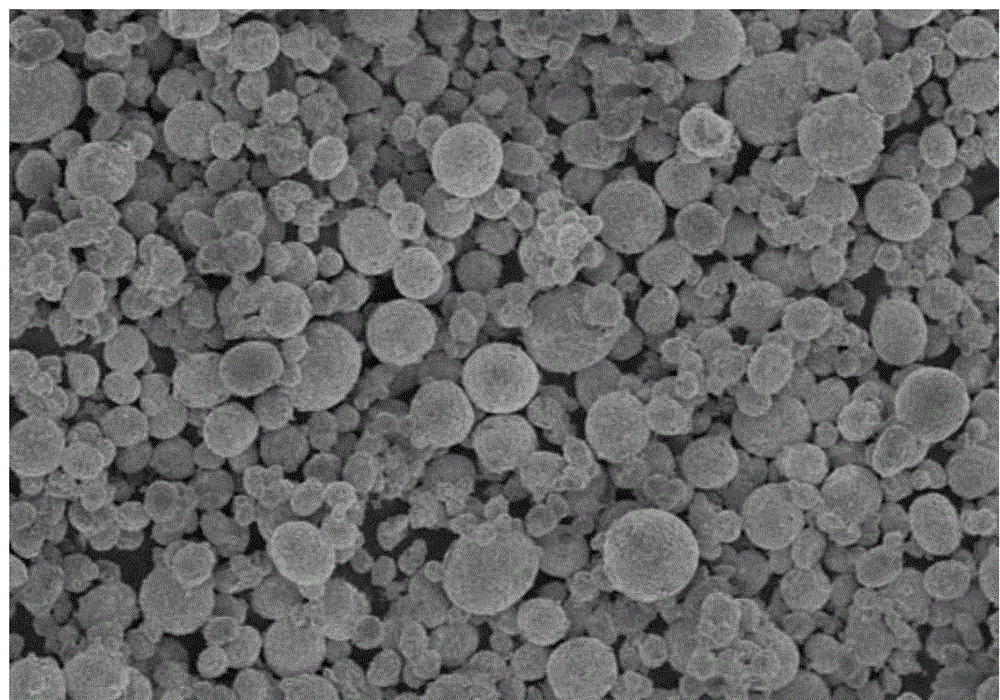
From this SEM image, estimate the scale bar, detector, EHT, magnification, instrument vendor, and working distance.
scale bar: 100000 nm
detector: SE(M)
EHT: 3 kV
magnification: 0.5 K X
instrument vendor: Hitachi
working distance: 8.7 mm